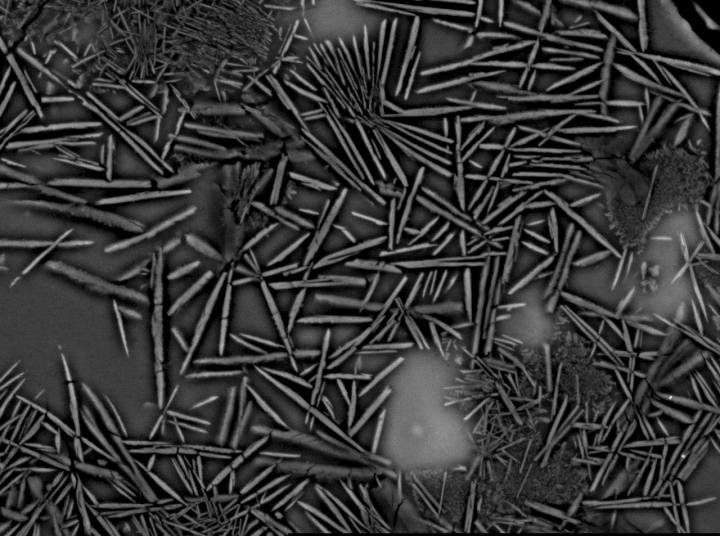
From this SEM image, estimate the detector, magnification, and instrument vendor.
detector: COMP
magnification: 1.9 K X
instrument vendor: JEOL